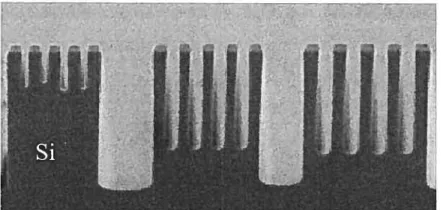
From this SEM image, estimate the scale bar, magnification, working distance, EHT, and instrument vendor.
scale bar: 1000 nm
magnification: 5 K X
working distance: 8 mm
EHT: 2 kV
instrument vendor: JEOL